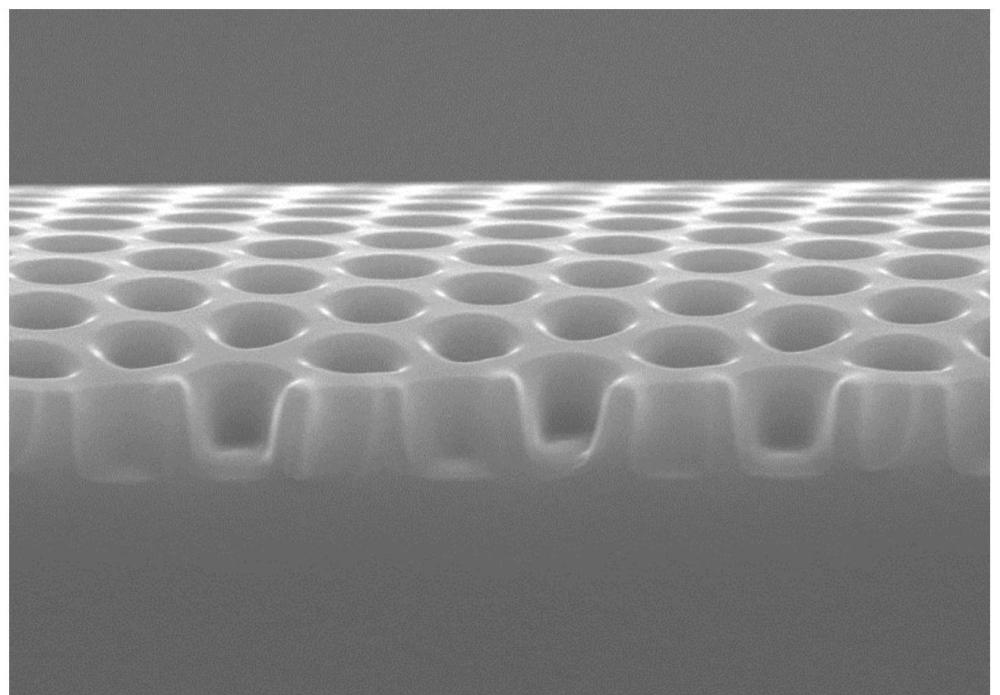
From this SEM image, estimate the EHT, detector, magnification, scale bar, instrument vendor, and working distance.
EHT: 15 kV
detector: SE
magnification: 5.48 K X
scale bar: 10000 nm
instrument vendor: Hitachi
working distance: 7.2 mm